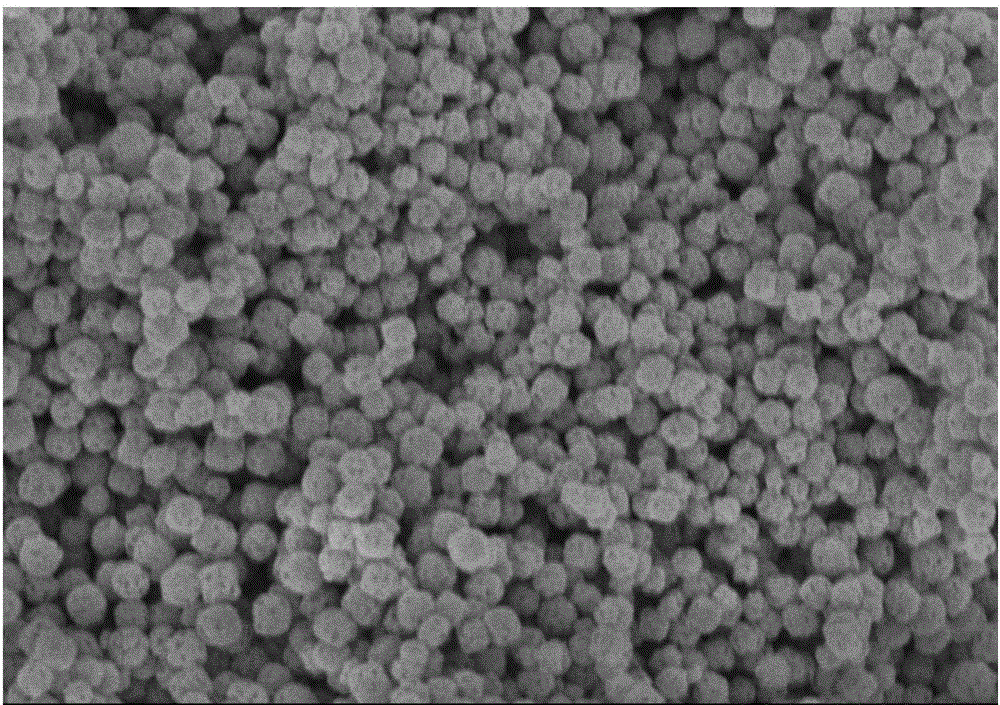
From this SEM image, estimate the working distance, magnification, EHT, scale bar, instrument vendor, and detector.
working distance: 8.7 mm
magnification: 30 K X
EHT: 3 kV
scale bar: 1000 nm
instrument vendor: Hitachi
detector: SE(U,LA2)